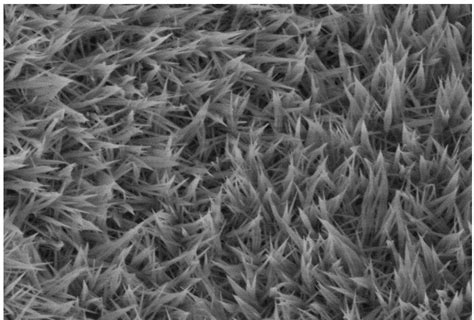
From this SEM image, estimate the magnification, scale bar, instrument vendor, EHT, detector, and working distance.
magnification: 20 K X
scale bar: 2000 nm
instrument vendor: Hitachi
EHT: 3 kV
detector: SE(UL)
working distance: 11 mm